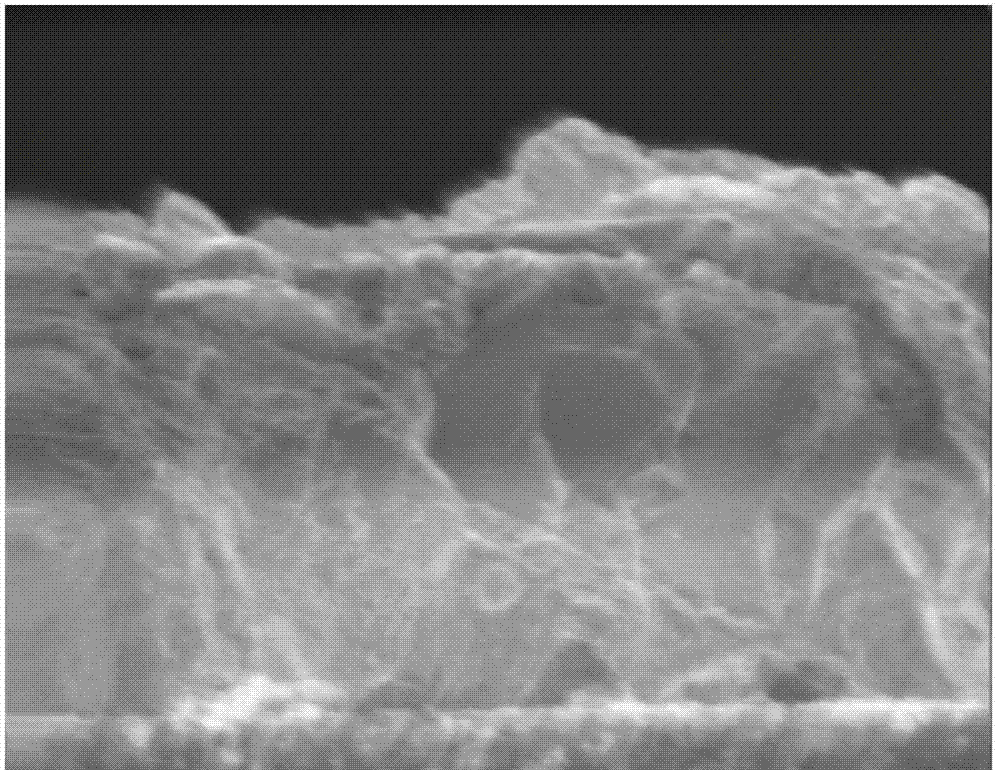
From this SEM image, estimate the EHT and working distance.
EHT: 5 kV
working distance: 37 mm